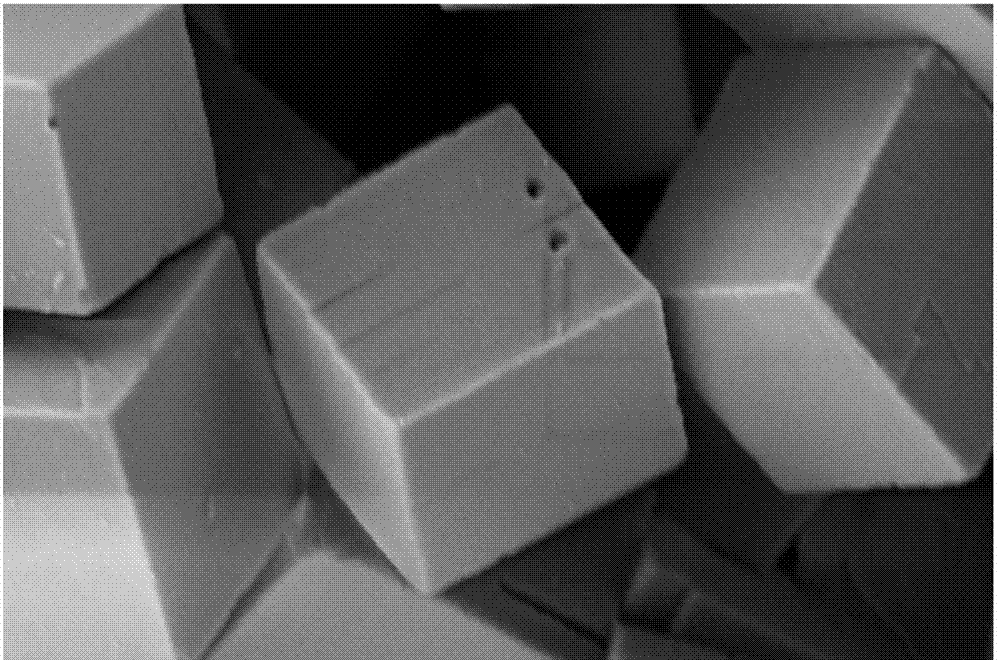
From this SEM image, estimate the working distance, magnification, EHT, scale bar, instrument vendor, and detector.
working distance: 5.5 mm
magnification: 20 K X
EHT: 2 kV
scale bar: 200 nm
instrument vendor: Zeiss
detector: SE2